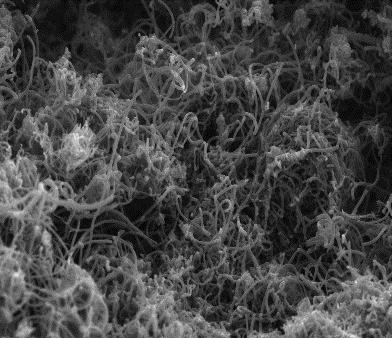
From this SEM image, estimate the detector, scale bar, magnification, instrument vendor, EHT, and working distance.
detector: InLens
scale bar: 1000 nm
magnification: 25 K X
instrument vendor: Zeiss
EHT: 5 kV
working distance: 3.2 mm